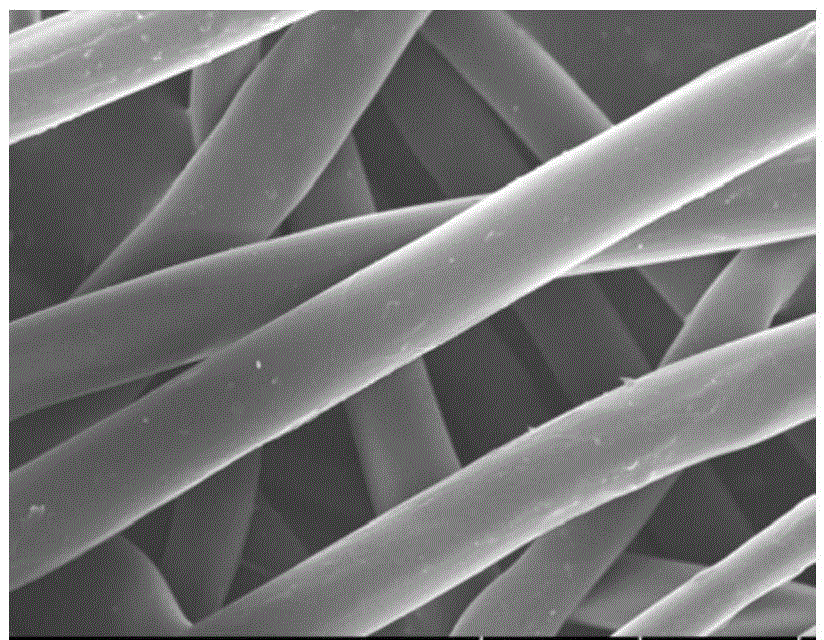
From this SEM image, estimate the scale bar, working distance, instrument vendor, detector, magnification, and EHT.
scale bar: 50000 nm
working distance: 8.3 mm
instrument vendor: Hitachi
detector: SE(UL)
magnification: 1 K X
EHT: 15 kV